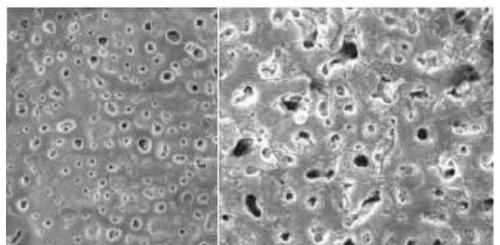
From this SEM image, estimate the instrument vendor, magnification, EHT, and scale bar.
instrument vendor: JEOL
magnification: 2 K X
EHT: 8 kV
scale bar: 10000 nm